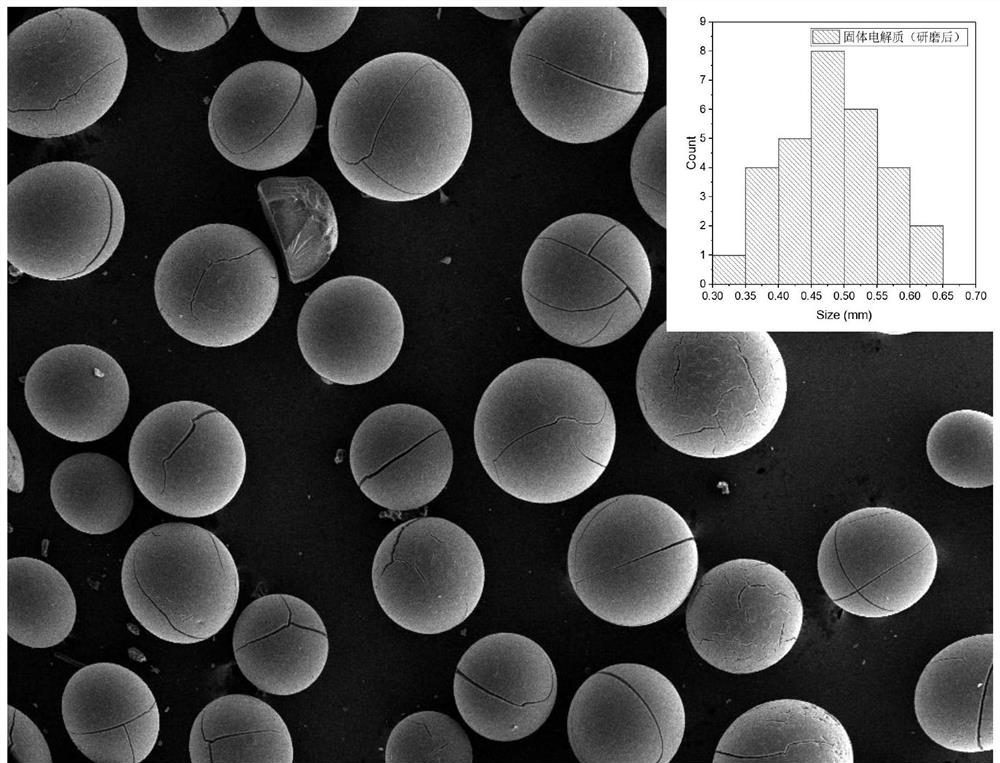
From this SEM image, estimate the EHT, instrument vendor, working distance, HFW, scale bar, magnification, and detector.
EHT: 20 kV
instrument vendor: other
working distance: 12 mm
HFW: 4220 µm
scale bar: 1000000 nm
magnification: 0.066 K X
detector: E-T